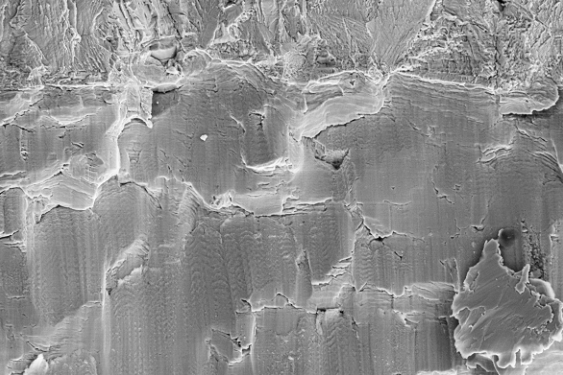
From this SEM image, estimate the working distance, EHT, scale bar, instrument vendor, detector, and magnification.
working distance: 15 mm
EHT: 20 kV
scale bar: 10000 nm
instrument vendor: Zeiss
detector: SE2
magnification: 0.5 K X